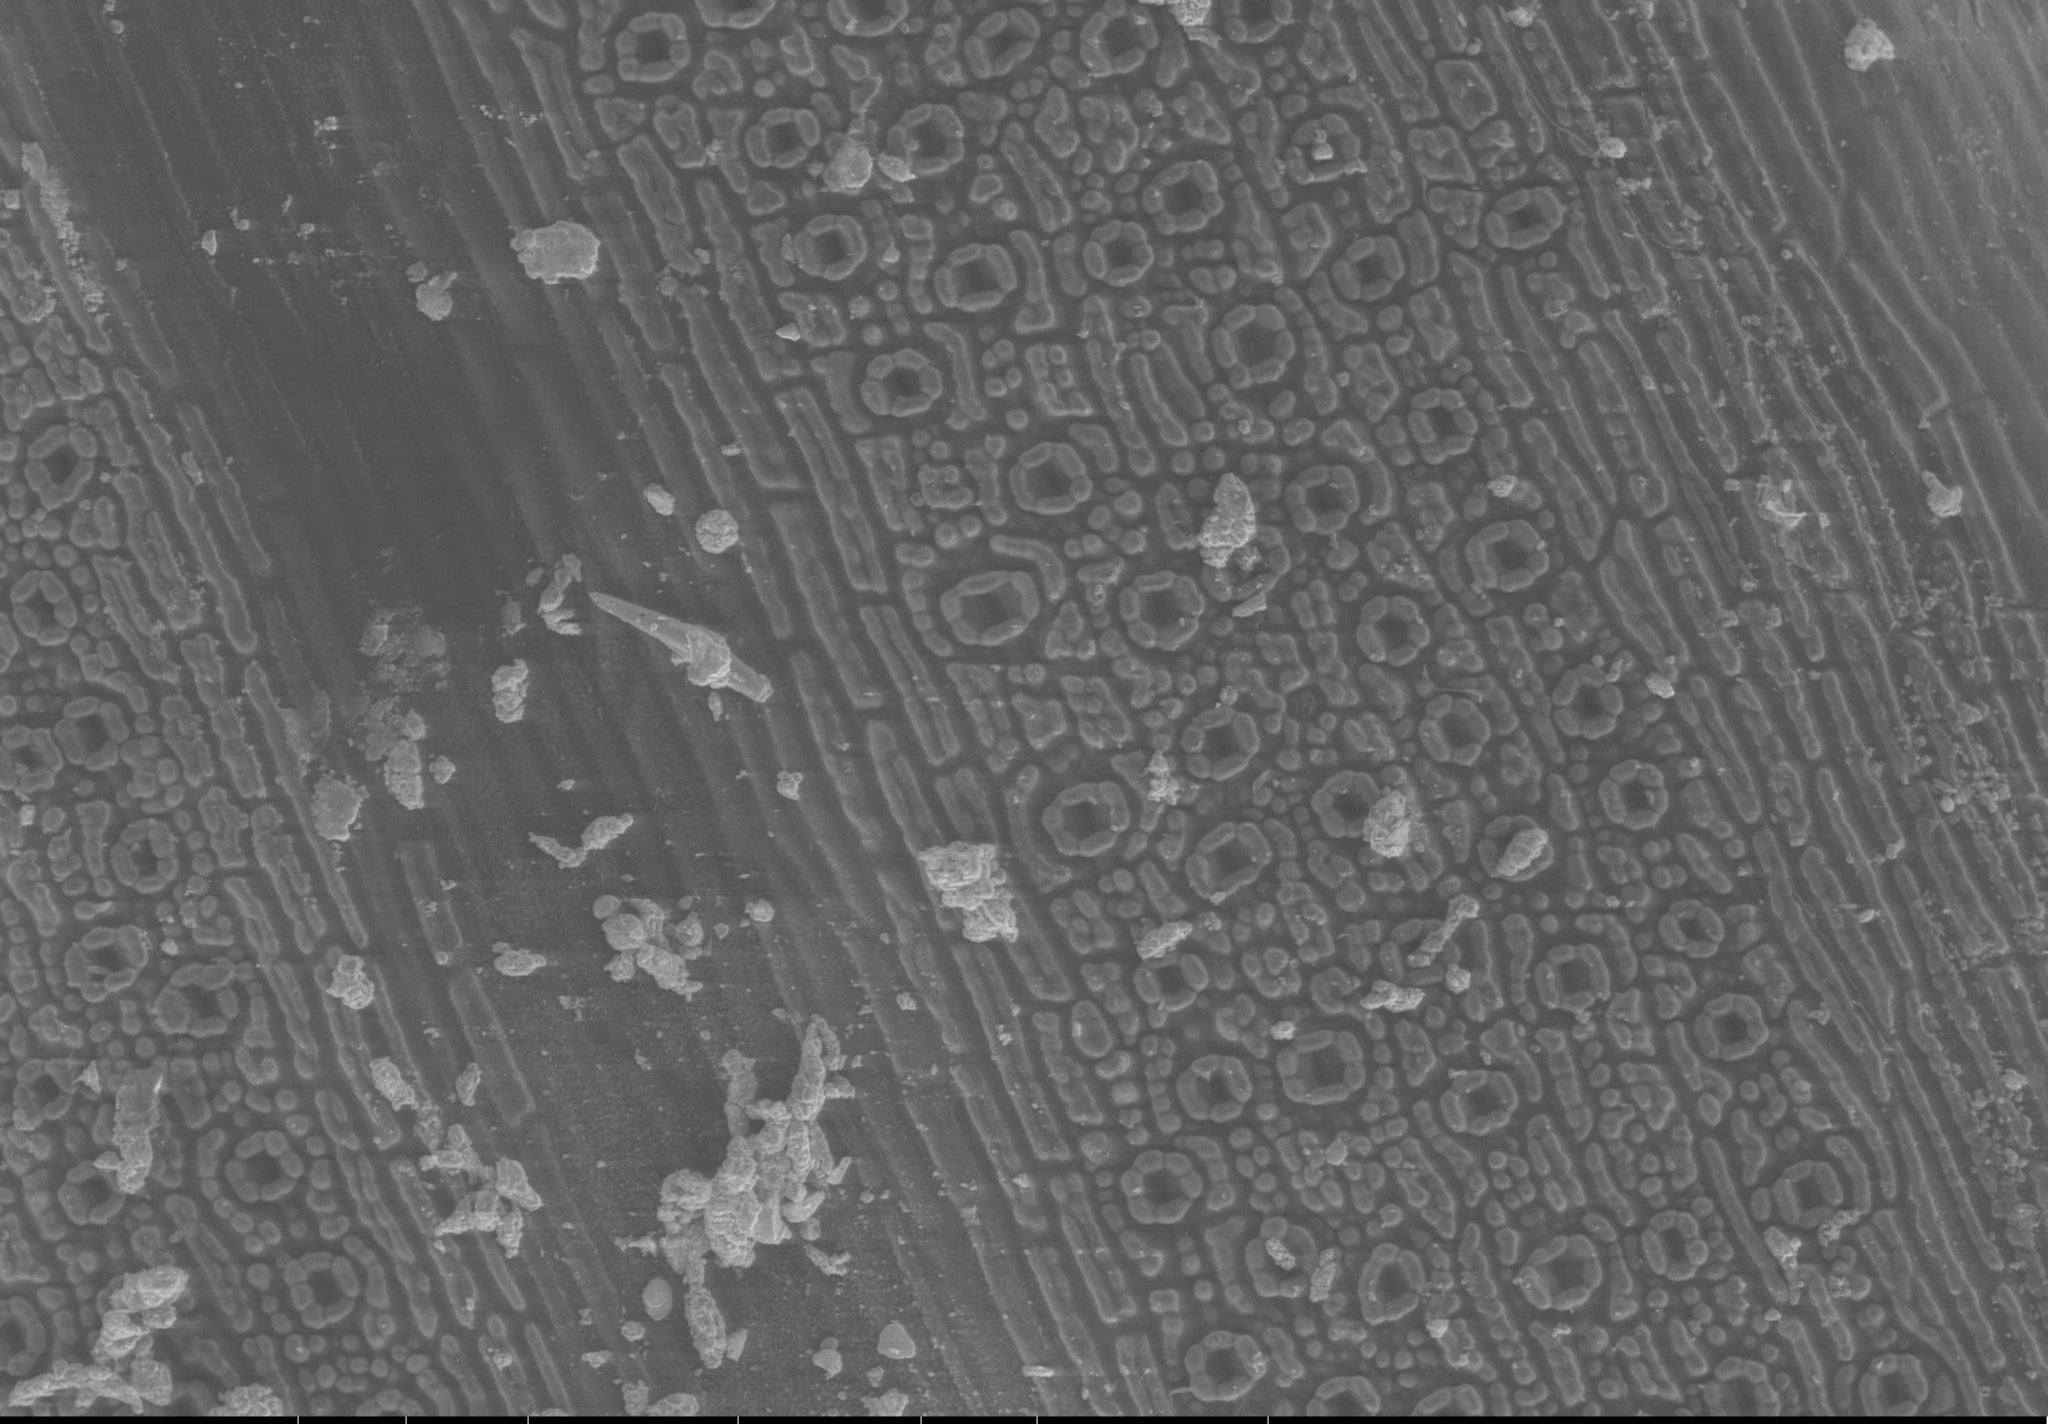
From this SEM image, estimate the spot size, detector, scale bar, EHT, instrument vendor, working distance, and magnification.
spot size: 4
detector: LFD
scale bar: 400000 nm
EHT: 12.5 kV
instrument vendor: FEI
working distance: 10.2 mm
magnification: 0.25 K X